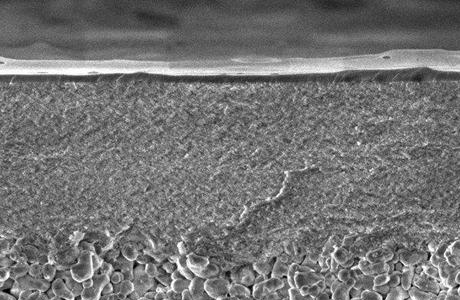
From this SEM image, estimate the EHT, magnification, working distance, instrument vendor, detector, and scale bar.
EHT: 2 kV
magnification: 50 K X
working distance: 7.2 mm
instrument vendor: Zeiss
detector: InLens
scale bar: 200 nm